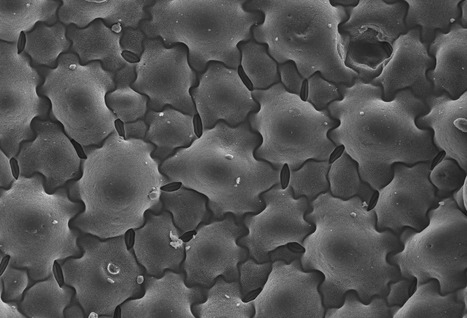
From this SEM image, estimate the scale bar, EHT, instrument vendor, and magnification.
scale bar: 200000 nm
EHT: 20 kV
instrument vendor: Tescan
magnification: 0.15 K X